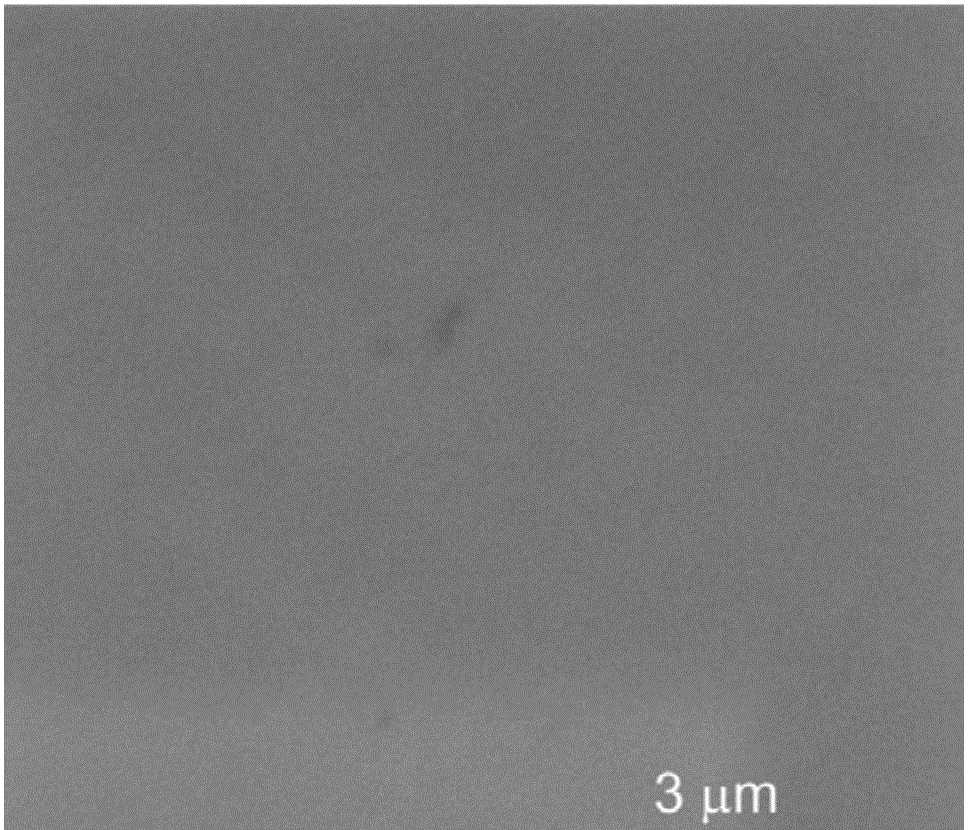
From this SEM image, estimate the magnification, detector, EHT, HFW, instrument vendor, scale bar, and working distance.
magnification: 20 K X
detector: ETD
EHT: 10 kV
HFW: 6.35 µm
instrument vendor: FEI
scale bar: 3000 nm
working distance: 9.7 mm